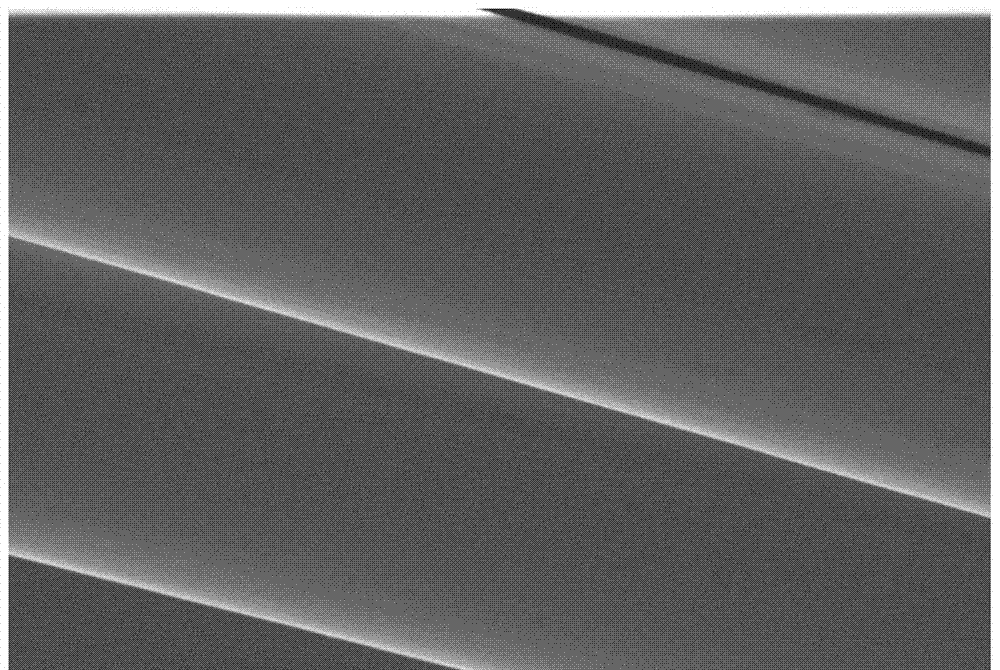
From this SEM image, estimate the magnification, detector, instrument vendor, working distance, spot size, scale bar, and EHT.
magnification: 10 K X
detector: ETD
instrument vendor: FEI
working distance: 2.1 mm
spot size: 2.5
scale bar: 10000 nm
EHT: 5 kV